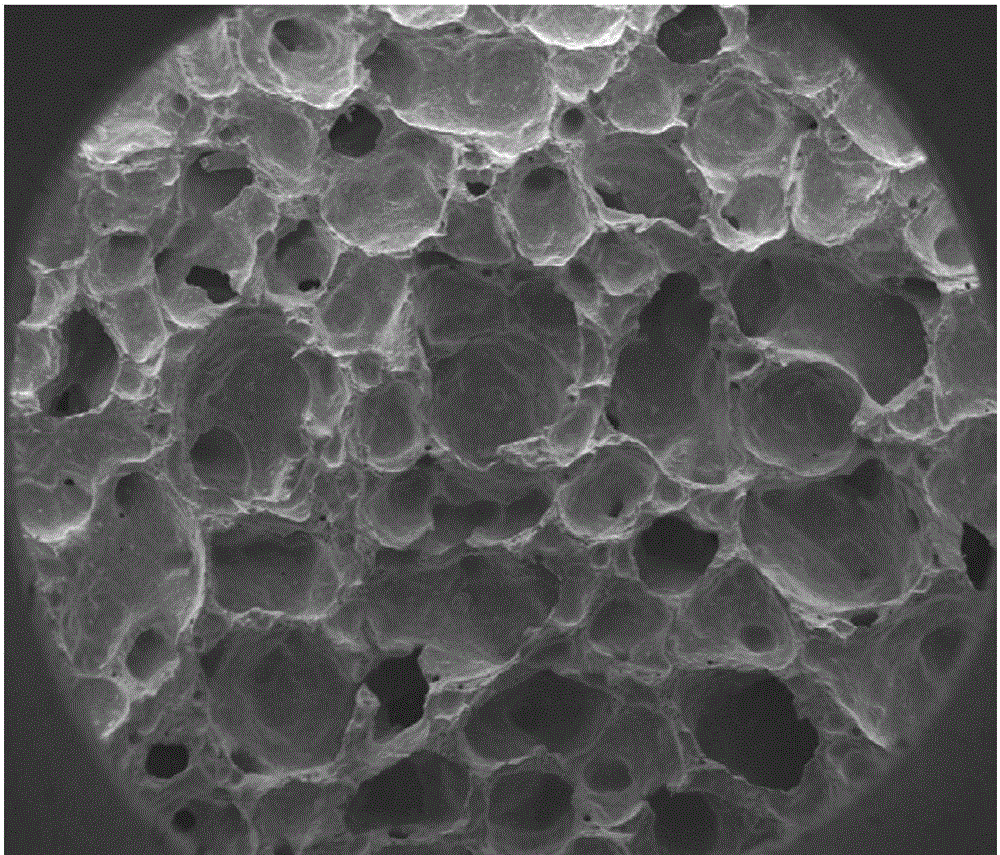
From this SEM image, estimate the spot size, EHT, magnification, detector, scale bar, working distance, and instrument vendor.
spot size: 3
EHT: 10 kV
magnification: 0.054 K X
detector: ETD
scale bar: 1000000 nm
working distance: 10.1 mm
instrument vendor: FEI